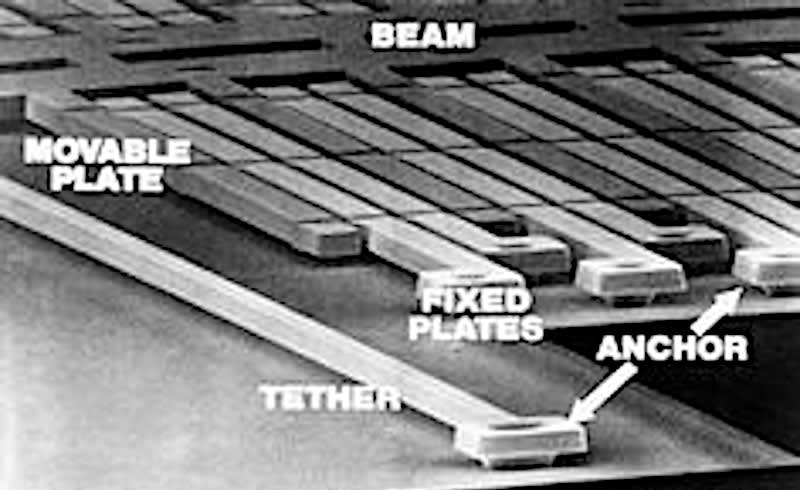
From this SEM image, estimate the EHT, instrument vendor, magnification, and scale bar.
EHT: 20 kV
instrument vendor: other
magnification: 1.5 K X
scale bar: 10000 nm